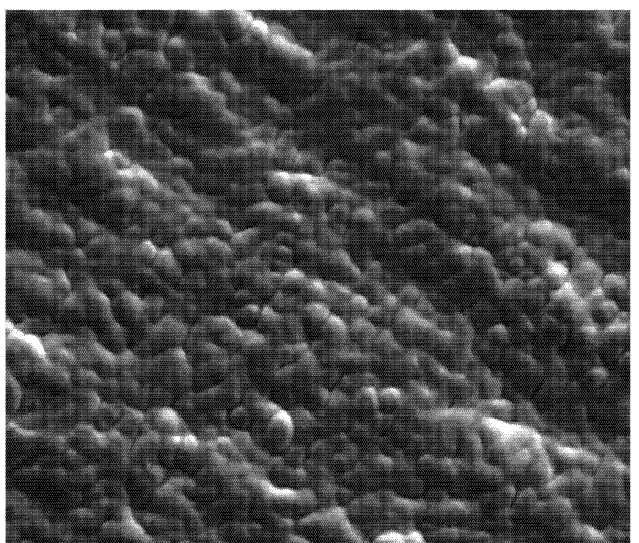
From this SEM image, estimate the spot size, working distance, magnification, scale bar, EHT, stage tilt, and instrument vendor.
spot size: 4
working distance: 9 mm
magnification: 5 K X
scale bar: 20000 nm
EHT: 10 kV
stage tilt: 0°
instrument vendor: FEI